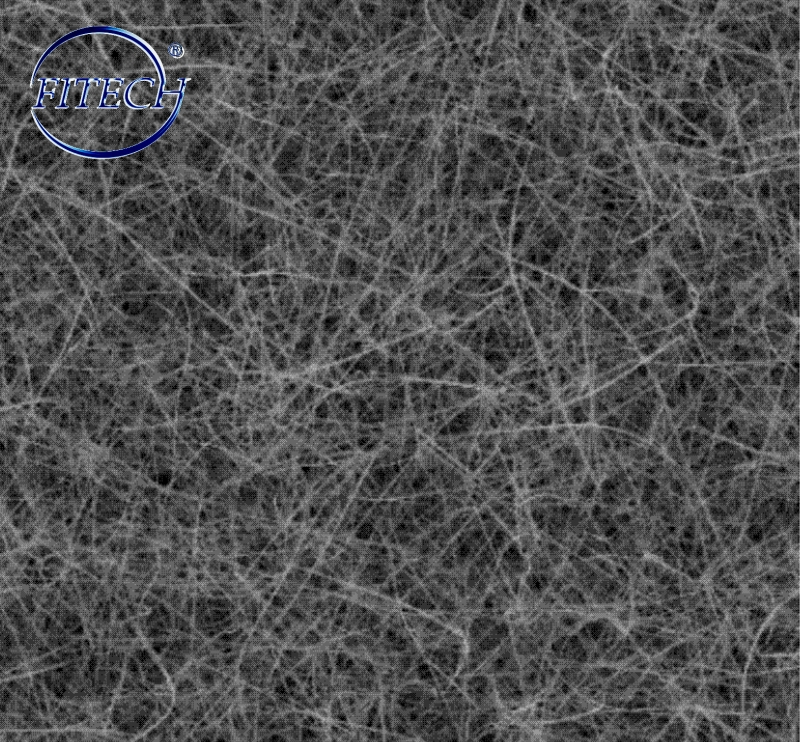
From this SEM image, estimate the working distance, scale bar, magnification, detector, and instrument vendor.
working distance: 12 mm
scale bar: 10000 nm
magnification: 5 K X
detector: SE(M)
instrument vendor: Hitachi